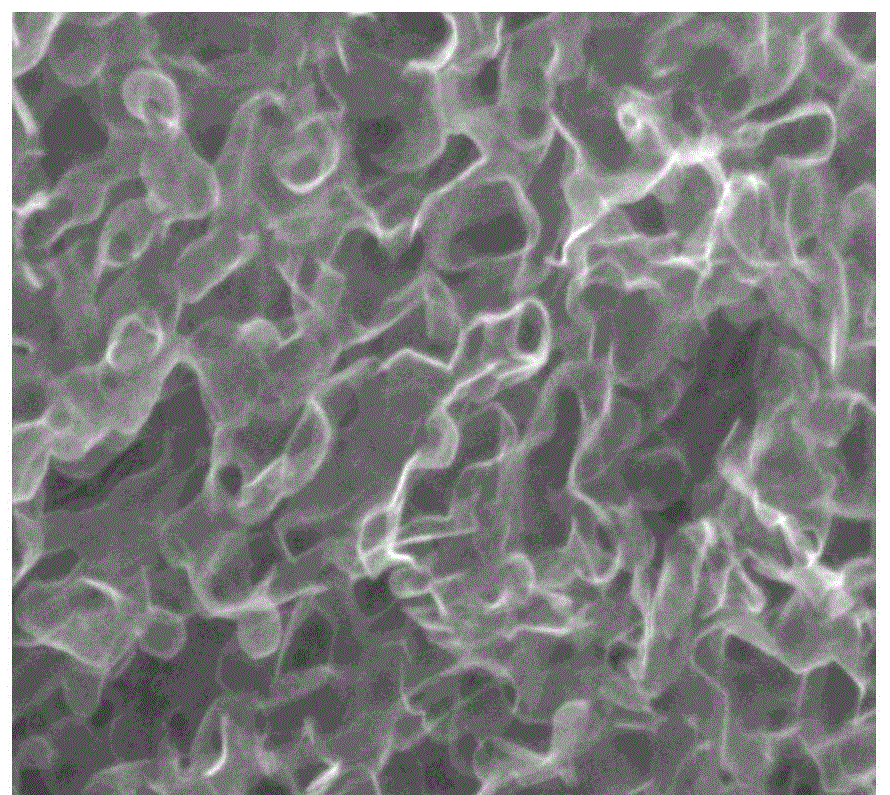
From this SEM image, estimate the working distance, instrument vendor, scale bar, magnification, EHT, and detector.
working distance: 9.2 mm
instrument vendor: Hitachi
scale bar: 300 nm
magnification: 150 K X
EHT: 5 kV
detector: SE(UL)